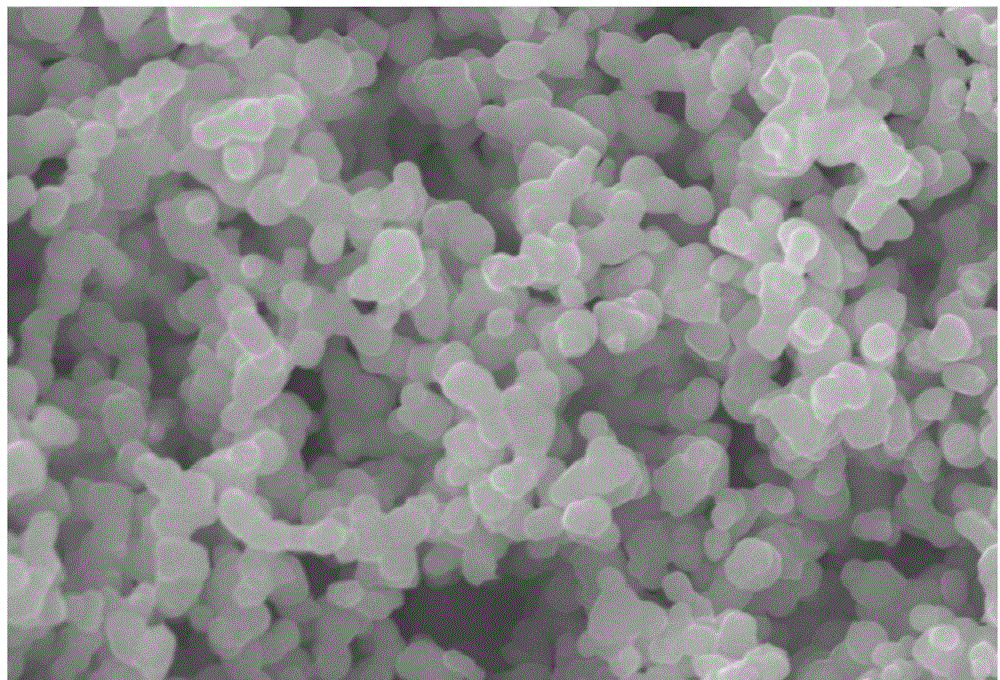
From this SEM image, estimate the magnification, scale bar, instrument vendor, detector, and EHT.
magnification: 5 K X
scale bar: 5000 nm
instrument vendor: JEOL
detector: SEI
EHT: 30 kV